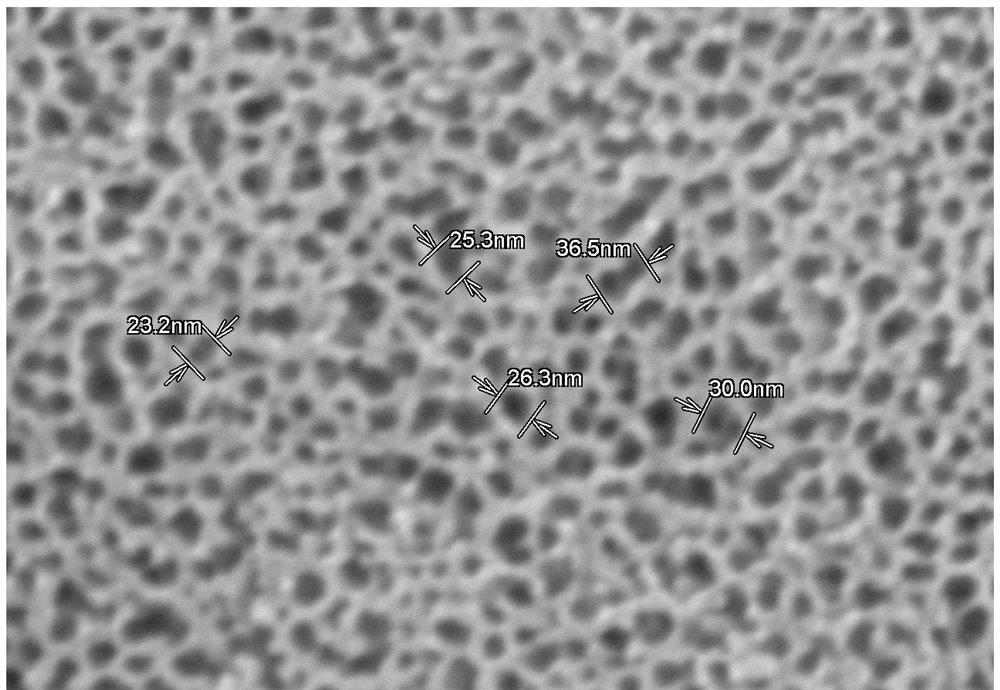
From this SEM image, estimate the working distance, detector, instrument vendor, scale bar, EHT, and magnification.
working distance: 4 mm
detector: SE(M)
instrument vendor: Hitachi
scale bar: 200 nm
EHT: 5 kV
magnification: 200 K X